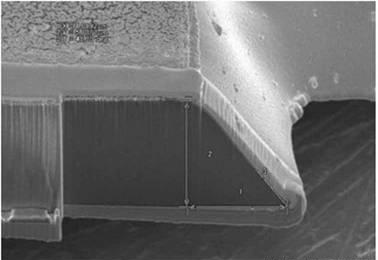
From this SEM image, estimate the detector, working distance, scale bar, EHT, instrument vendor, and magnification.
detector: SE(M)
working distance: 16.4 mm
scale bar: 5000 nm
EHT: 3 kV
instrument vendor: Hitachi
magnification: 7 K X